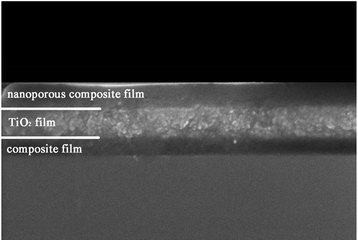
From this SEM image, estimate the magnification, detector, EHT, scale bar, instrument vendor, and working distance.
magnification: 100 K X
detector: SE(U,LA0)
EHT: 5 kV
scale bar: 500 nm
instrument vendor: Hitachi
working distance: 9 mm